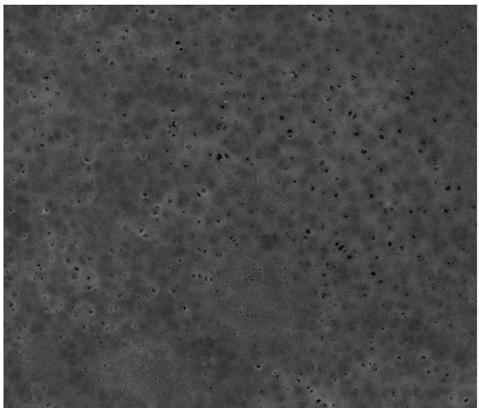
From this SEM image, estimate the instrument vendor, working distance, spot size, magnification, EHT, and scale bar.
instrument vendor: FEI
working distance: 12.9 mm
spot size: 3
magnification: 5 K X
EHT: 20 kV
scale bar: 20000 nm